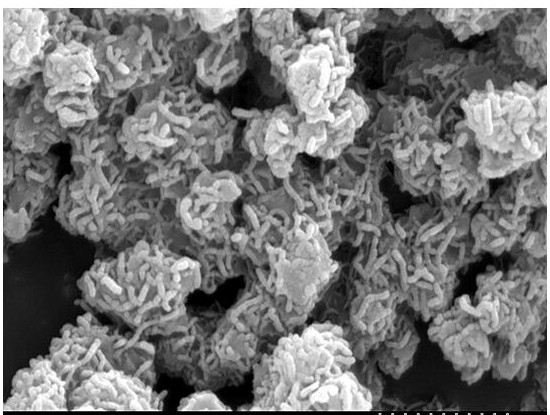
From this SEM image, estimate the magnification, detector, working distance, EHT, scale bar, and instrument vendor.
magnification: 30 K X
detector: SE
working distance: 17.3 mm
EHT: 20 kV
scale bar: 1000 nm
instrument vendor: Hitachi